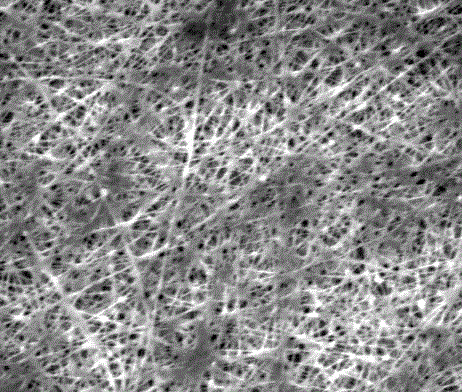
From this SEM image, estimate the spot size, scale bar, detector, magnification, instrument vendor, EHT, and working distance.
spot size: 3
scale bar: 10000 nm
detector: ETD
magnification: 8 K X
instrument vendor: FEI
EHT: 10 kV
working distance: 14.3 mm